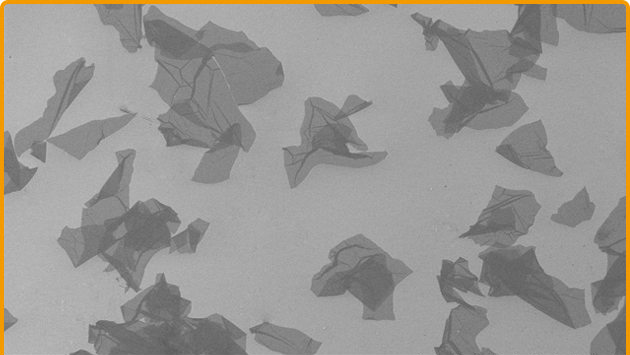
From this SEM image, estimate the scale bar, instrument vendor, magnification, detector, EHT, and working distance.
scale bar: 100000 nm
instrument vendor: Hitachi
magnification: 0.4 K X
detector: SE(M)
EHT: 3 kV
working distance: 9.4 mm